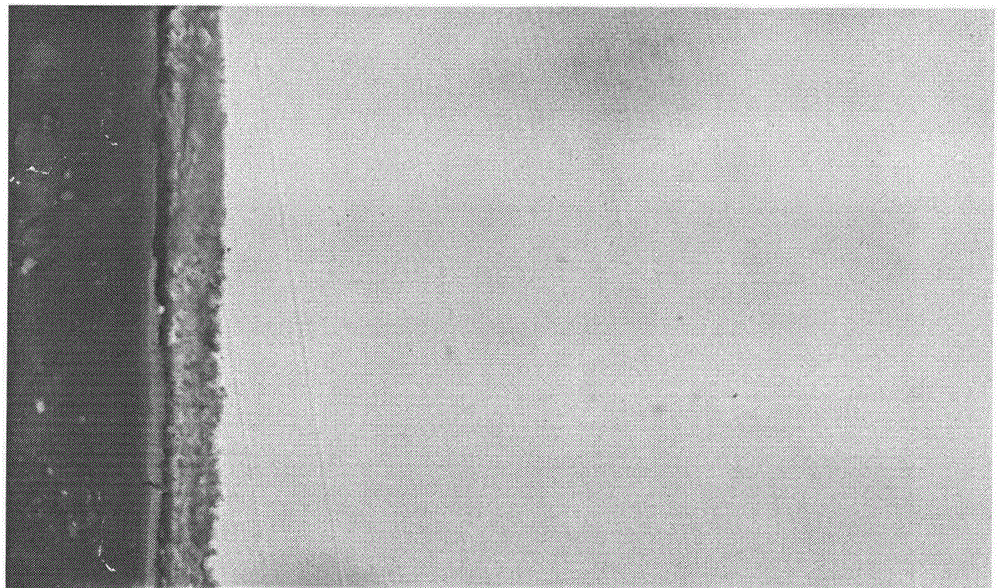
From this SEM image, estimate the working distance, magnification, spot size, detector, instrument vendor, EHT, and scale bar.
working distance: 7.4 mm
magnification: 4 K X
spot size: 5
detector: BSE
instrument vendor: FEI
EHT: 15 kV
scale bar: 5000 nm